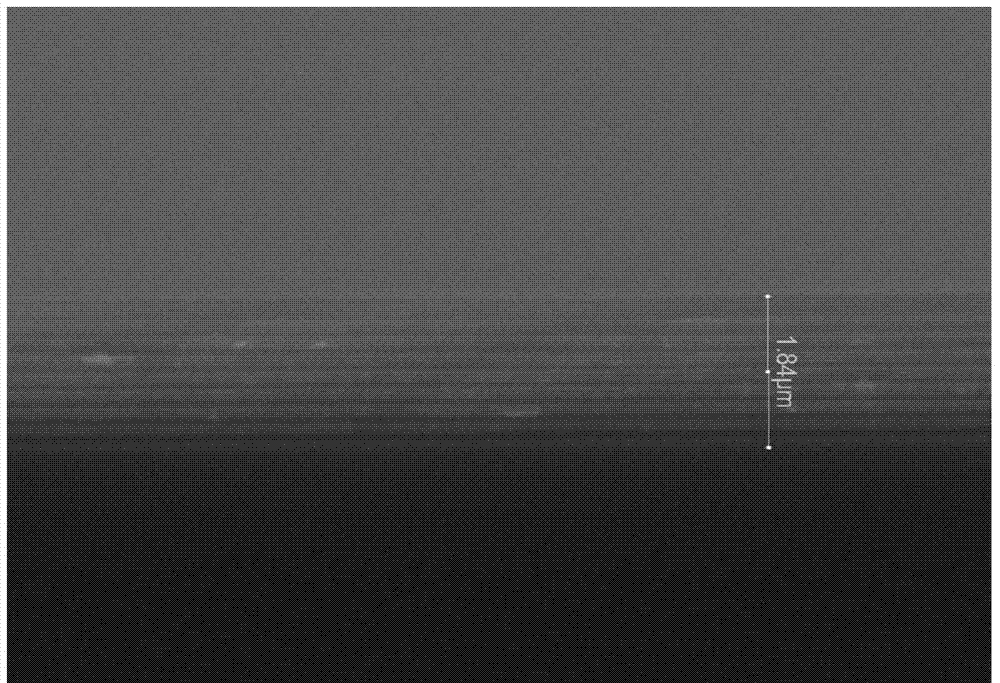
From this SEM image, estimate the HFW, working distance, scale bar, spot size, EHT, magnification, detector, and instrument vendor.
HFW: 9.59 µm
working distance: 4.9 mm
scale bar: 4000 nm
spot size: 3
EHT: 20 kV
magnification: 31.12 K X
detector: ETD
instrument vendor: FEI